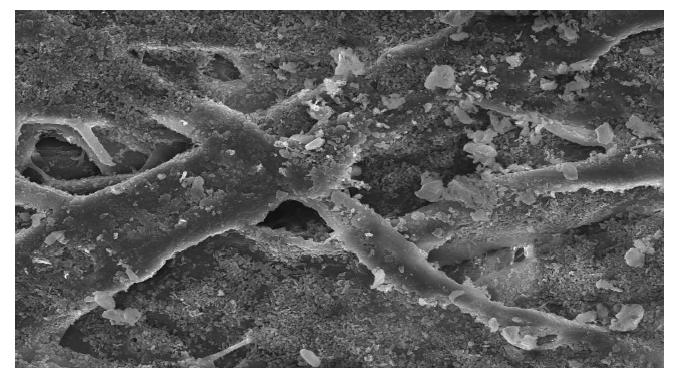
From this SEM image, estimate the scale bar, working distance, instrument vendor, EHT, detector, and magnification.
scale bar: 50000 nm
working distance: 12 mm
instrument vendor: Hitachi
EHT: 15 kV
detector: SE(U)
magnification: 1 K X